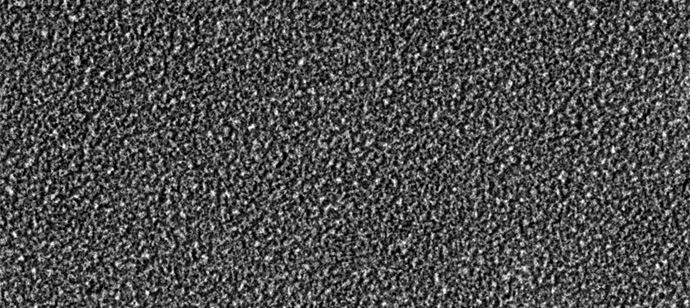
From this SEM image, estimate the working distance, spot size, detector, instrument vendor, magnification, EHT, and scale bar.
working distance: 19 mm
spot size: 50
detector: SEI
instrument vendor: JEOL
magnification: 2 K X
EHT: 15 kV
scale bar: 10000 nm